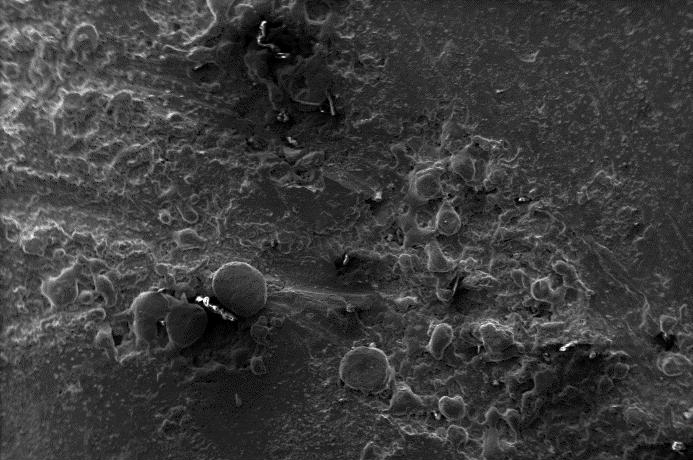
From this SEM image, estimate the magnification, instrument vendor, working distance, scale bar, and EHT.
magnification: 0.15 K X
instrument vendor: Zeiss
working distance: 8.5 mm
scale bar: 100000 nm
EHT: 10 kV